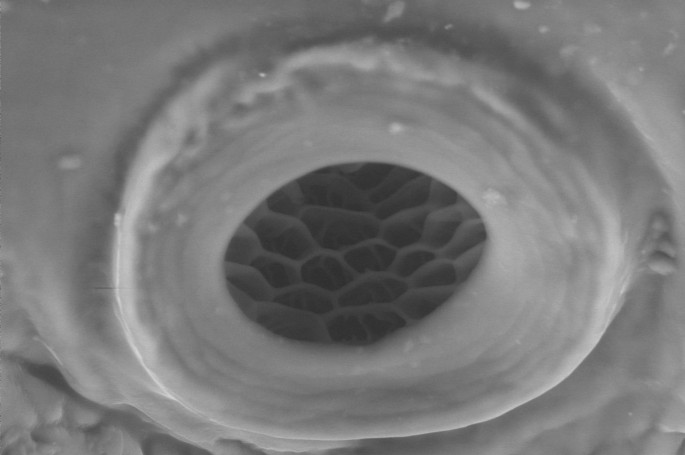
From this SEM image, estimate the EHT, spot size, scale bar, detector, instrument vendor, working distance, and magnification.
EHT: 10 kV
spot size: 4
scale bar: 10000 nm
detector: GSE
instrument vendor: FEI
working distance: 5 mm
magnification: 1.971 K X